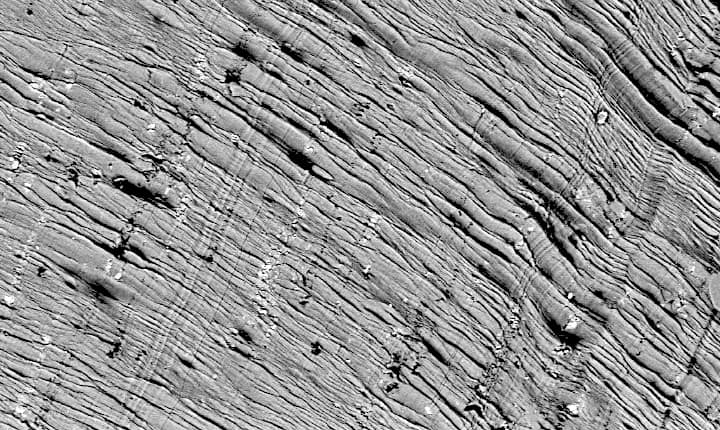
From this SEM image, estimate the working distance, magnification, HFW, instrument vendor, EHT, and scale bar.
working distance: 10.773 mm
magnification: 0.68 K X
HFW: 395 µm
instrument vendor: Phenom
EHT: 15 kV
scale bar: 100000 nm